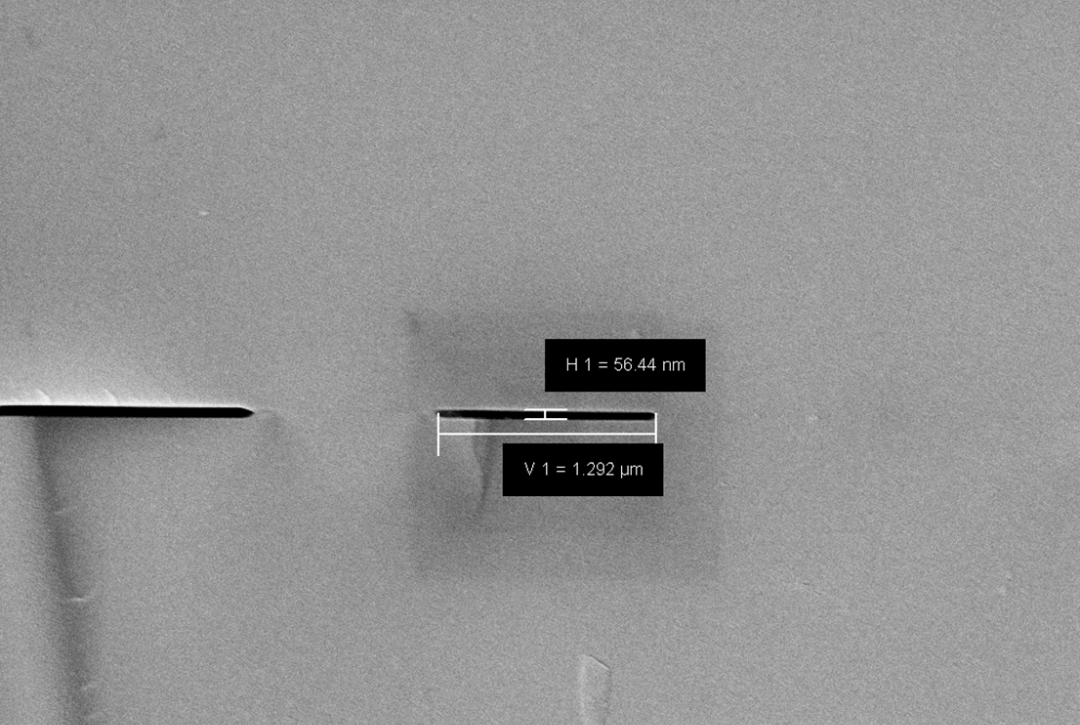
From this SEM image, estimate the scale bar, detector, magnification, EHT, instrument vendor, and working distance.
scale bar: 1000 nm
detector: SE2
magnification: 17.81 K X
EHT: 12 kV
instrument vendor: Zeiss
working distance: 16.6 mm